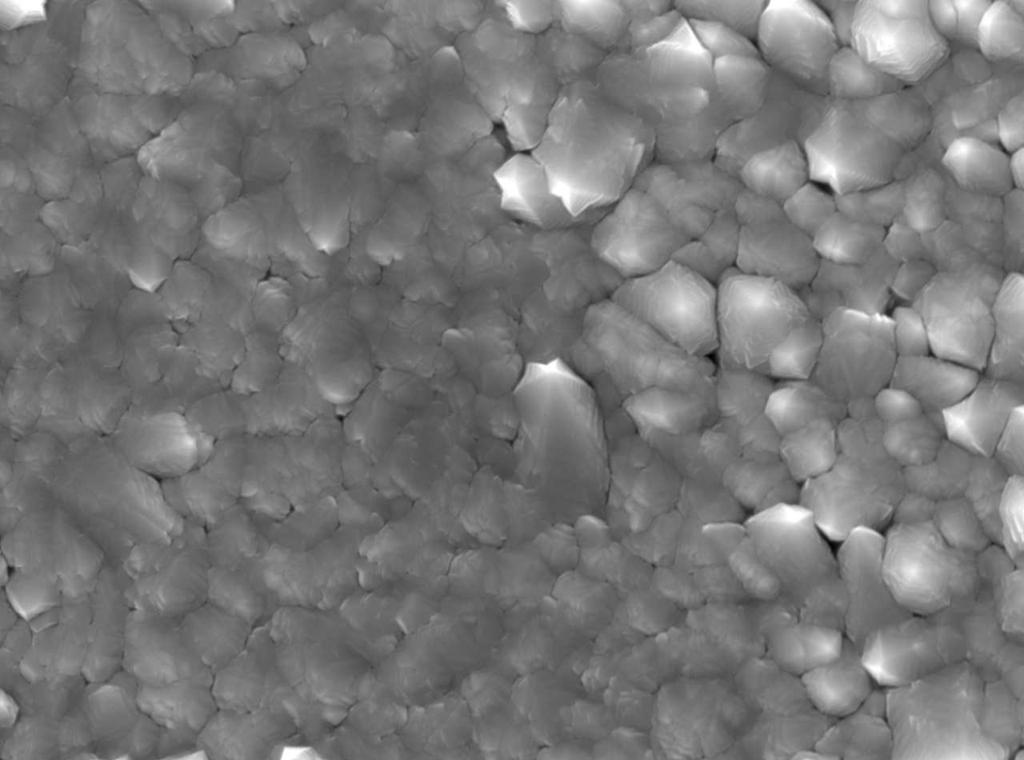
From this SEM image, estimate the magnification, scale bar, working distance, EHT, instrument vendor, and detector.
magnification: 10 K X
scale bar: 1000 nm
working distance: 9.8 mm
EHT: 15 kV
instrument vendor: JEOL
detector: SEI